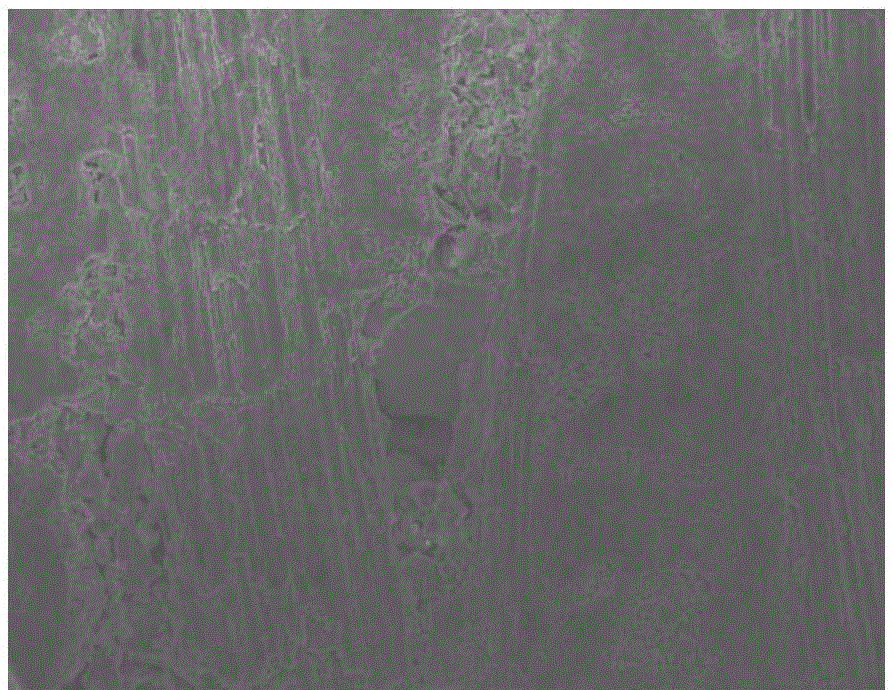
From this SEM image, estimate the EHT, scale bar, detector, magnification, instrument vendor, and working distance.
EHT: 15 kV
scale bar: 100000 nm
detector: SE(U)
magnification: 0.3 K X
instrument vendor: Hitachi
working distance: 9.5 mm